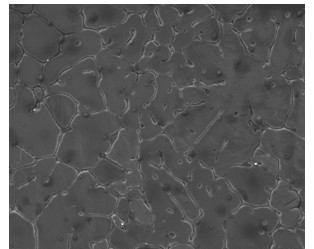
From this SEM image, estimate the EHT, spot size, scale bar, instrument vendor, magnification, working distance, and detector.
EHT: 15 kV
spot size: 5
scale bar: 50000 nm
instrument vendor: FEI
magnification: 1 K X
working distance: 10 mm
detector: ETD